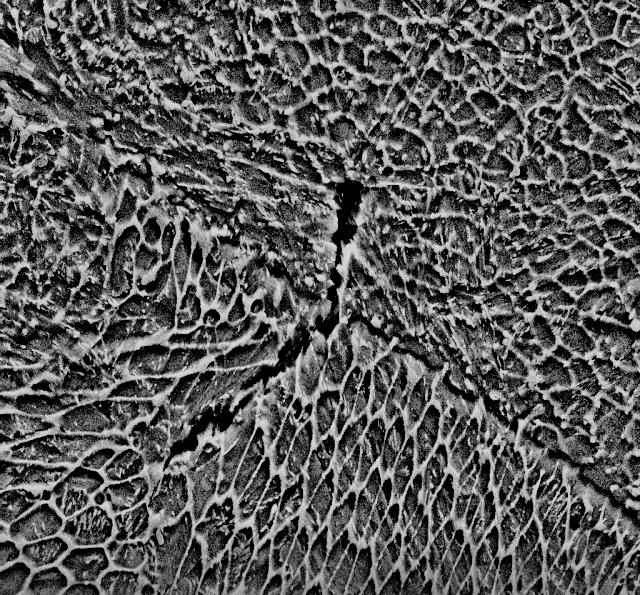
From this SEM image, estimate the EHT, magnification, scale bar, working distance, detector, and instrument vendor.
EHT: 15 kV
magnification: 10 K X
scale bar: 3000 nm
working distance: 8.3 mm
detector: SE2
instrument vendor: Zeiss